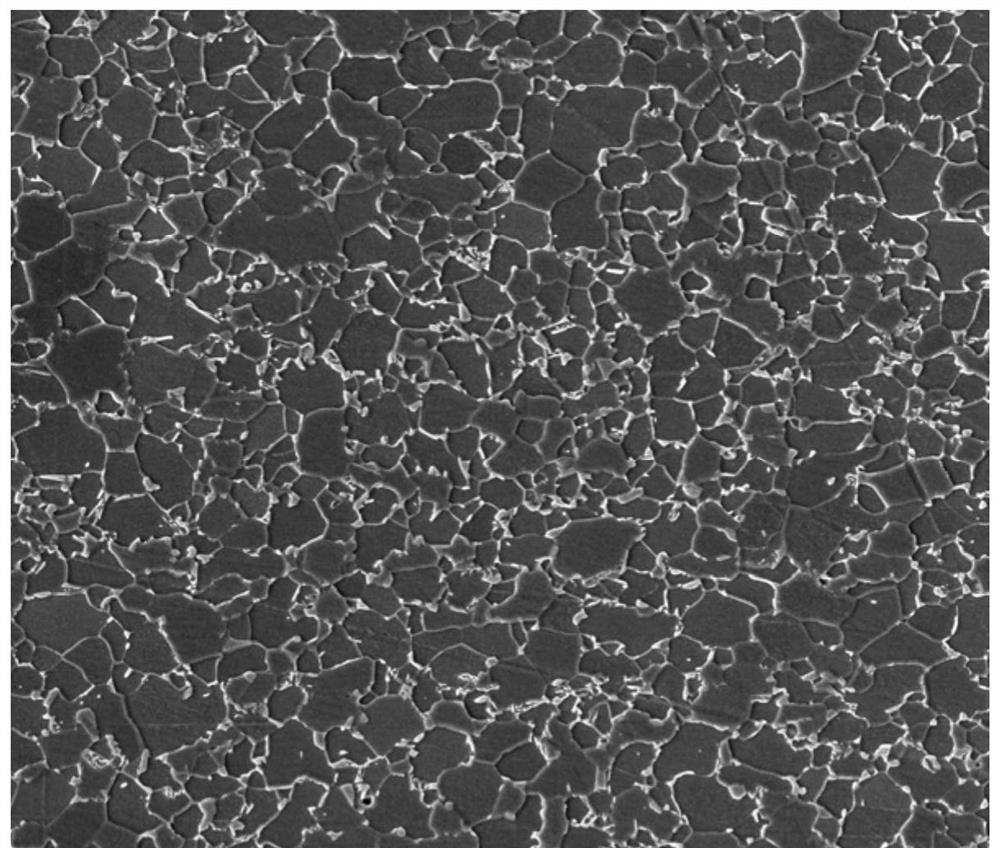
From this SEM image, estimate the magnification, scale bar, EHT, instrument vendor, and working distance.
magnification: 2 K X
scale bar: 100000 nm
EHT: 20 kV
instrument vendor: FEI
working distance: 11.7 mm